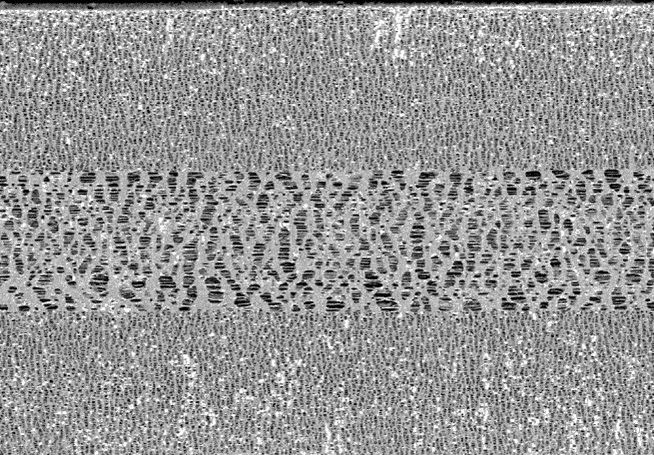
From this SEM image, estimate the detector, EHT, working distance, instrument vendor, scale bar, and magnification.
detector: LA100(U)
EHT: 0.7 kV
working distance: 3.2 mm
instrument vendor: Hitachi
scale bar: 10000 nm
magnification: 3.5 K X